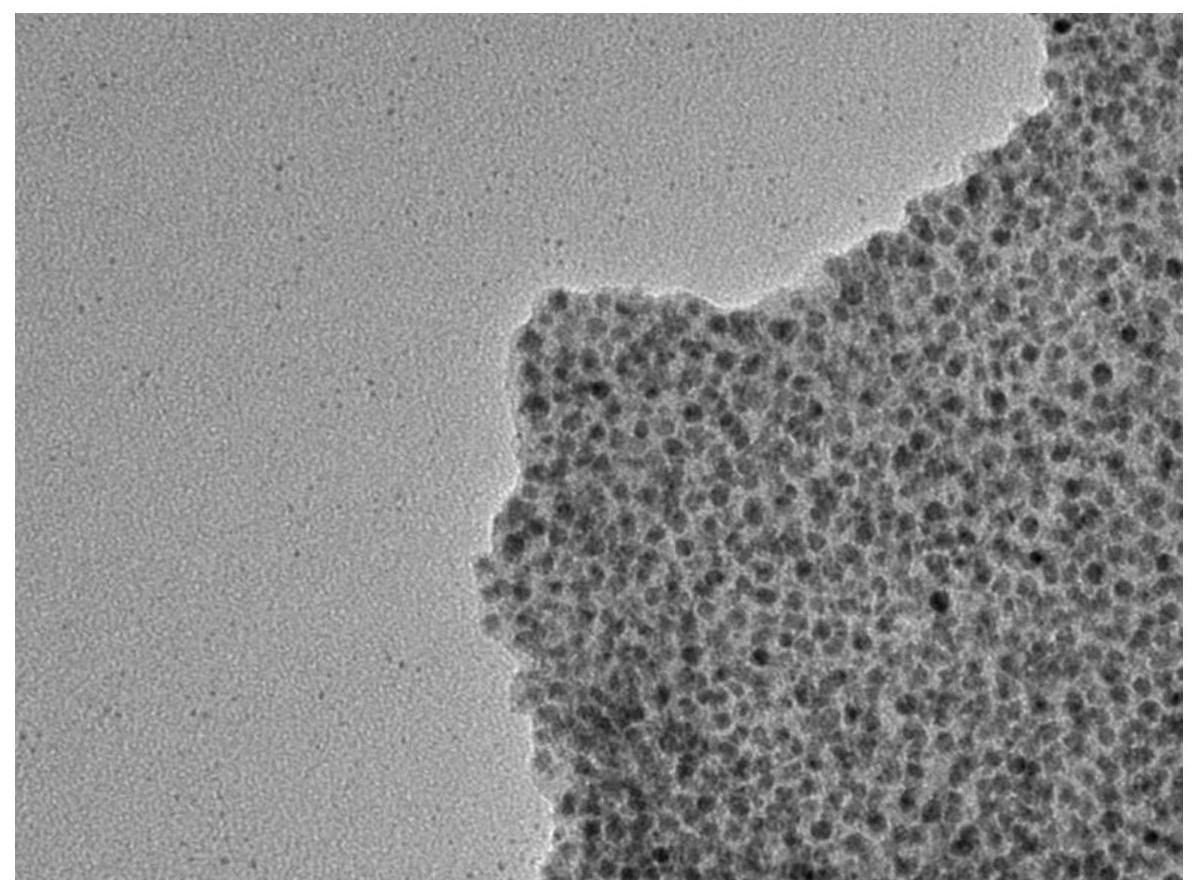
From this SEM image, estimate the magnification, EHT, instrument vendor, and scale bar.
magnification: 100 K X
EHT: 100 kV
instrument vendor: Hitachi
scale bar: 100 nm